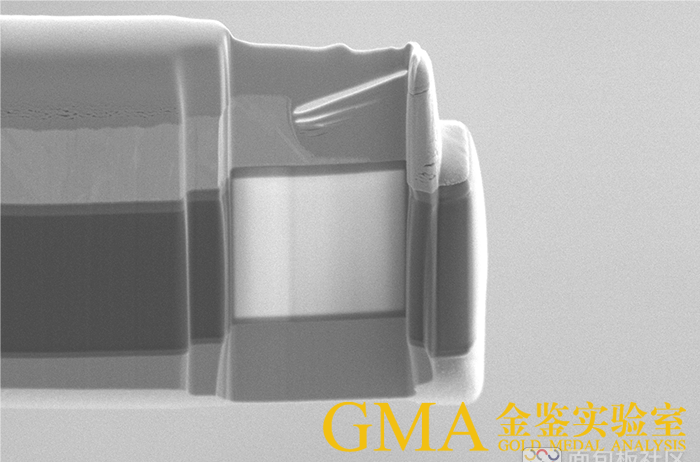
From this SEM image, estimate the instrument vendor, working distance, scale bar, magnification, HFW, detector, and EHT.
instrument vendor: FEI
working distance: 7 mm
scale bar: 5000 nm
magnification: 6.5 K X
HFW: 19.5 µm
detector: ETD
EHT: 3 kV